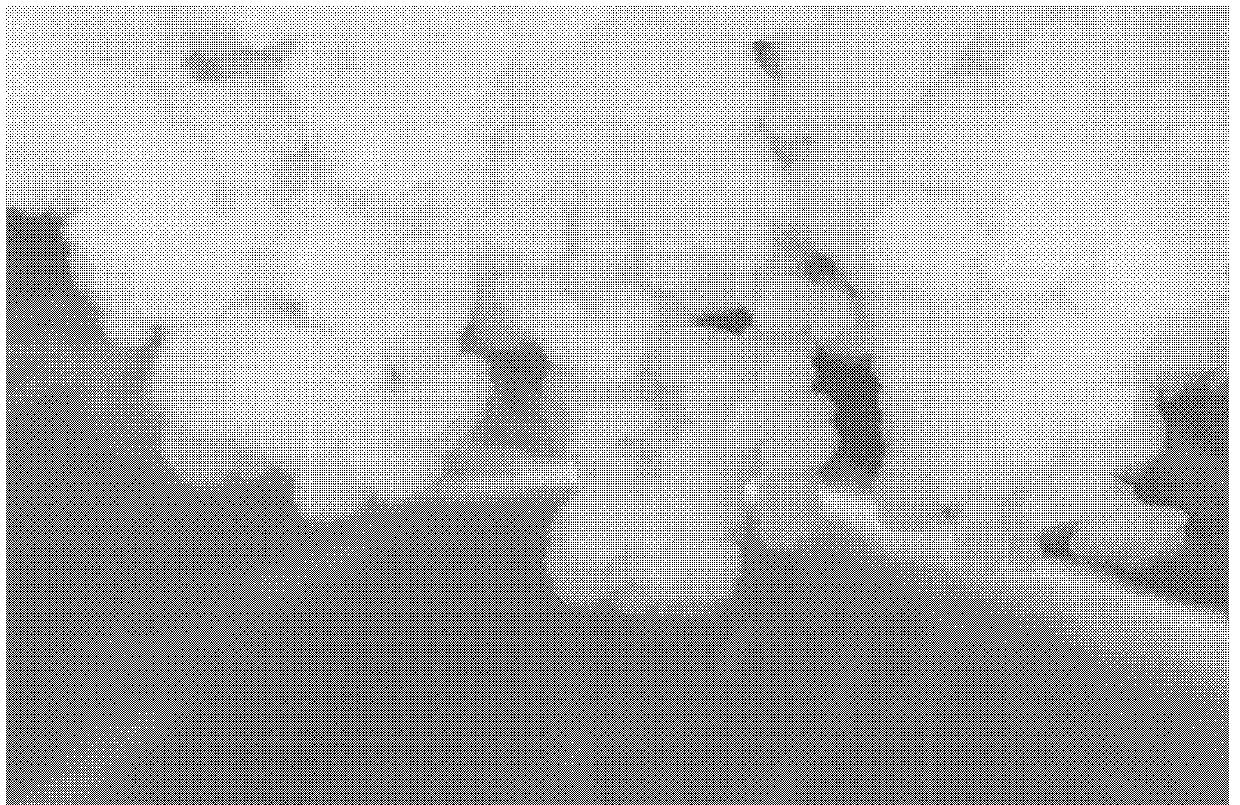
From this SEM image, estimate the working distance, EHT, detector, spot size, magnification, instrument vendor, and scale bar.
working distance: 4.4 mm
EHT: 15 kV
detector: TLD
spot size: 4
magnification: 100 K X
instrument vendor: FEI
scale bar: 200 nm